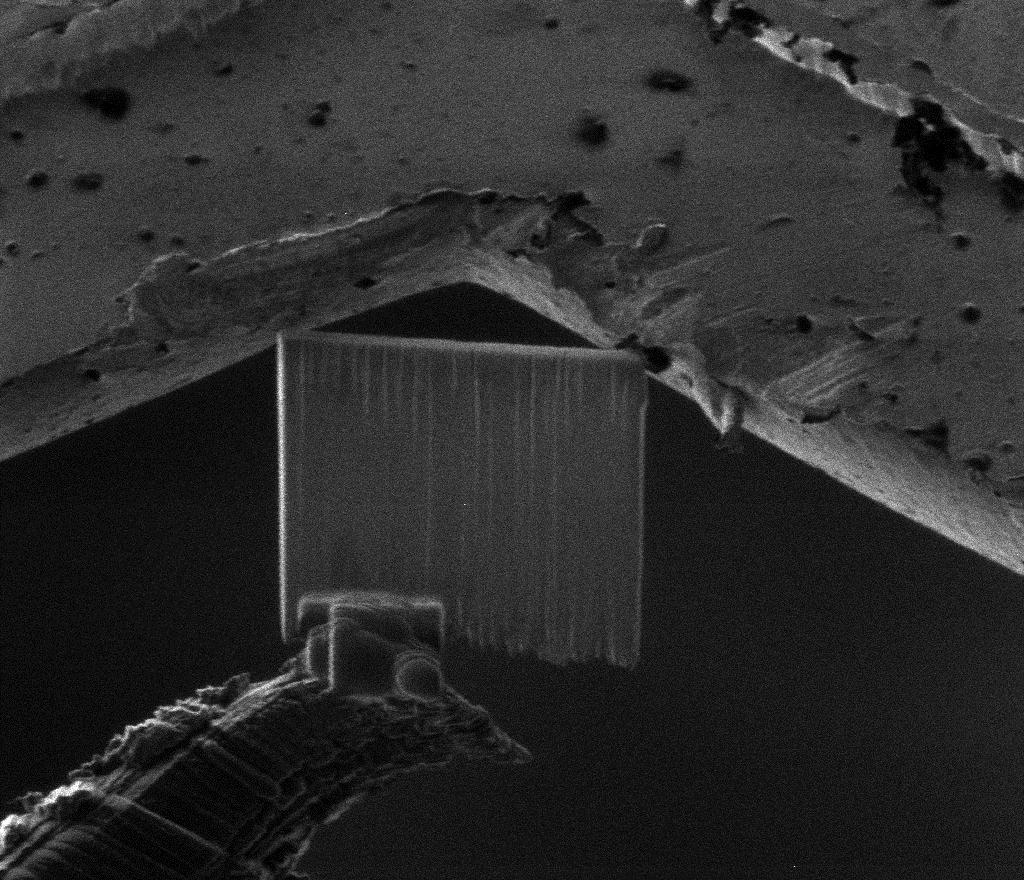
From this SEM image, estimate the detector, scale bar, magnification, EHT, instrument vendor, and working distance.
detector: CDEM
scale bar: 10000 nm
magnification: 3 K X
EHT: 30 kV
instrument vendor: FEI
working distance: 18.9 mm